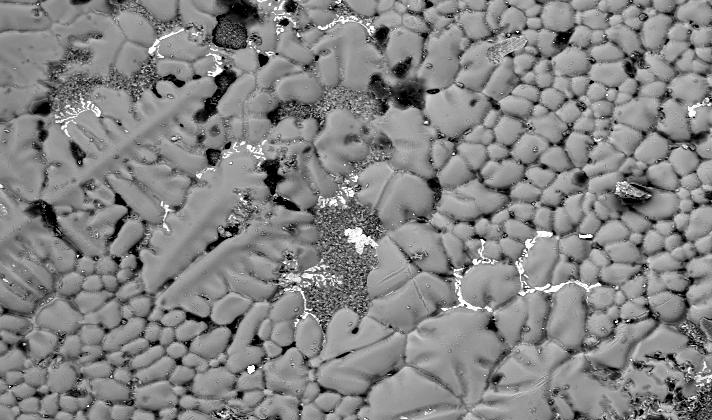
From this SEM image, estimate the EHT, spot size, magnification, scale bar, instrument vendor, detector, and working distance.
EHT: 20 kV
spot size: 6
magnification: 0.125 K X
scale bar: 200000 nm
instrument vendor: FEI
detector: BSE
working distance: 10 mm